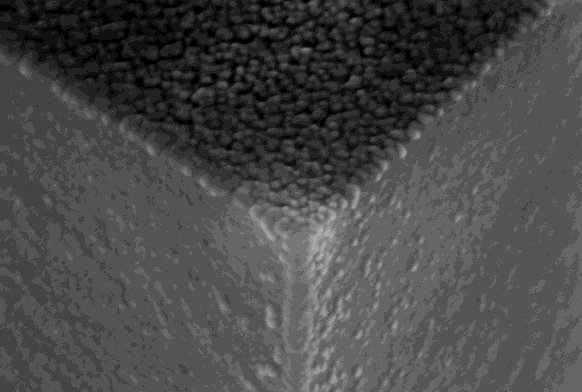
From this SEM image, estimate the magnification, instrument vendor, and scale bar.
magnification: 75 K X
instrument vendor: Zeiss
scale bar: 100 nm